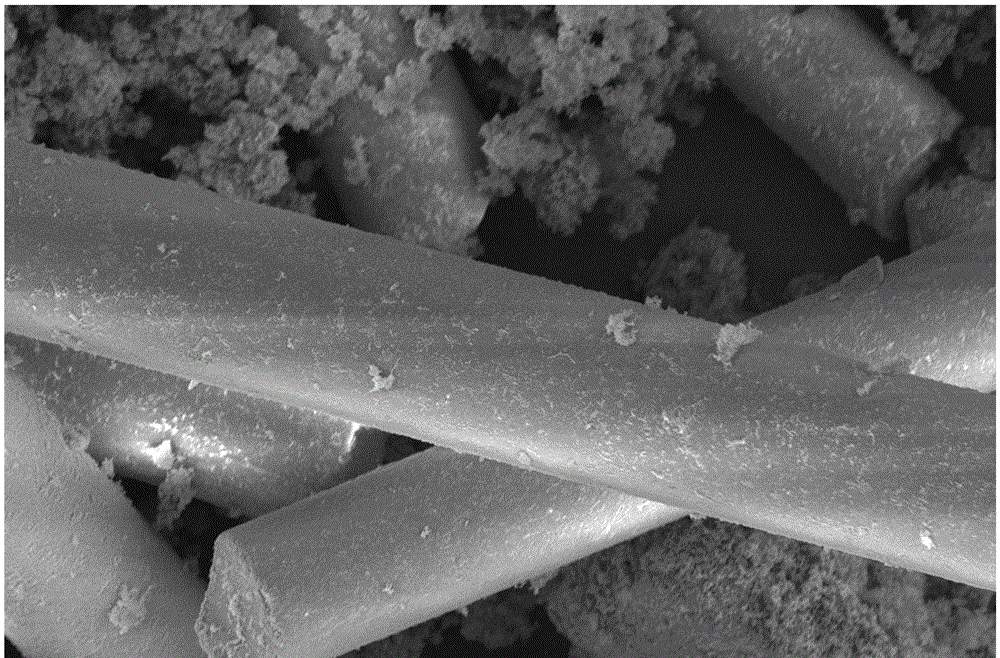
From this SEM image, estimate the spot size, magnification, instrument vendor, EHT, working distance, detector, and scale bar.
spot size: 3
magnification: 5 K X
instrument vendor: FEI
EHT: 5 kV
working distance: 5.2 mm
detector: ETD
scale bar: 30000 nm